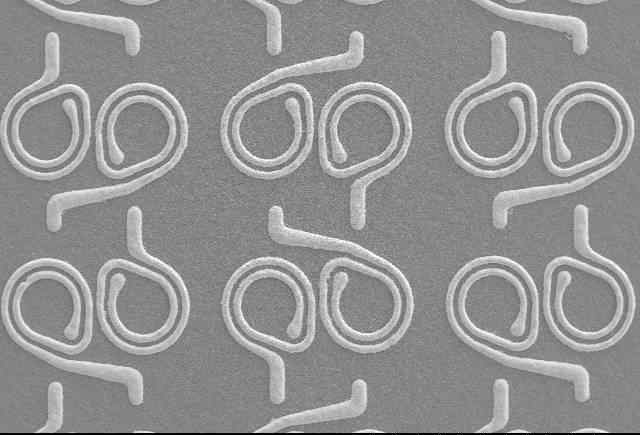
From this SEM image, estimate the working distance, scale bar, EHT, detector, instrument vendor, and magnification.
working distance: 13.1 mm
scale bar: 1000000 nm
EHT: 20 kV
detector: SE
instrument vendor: other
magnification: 0.045 K X